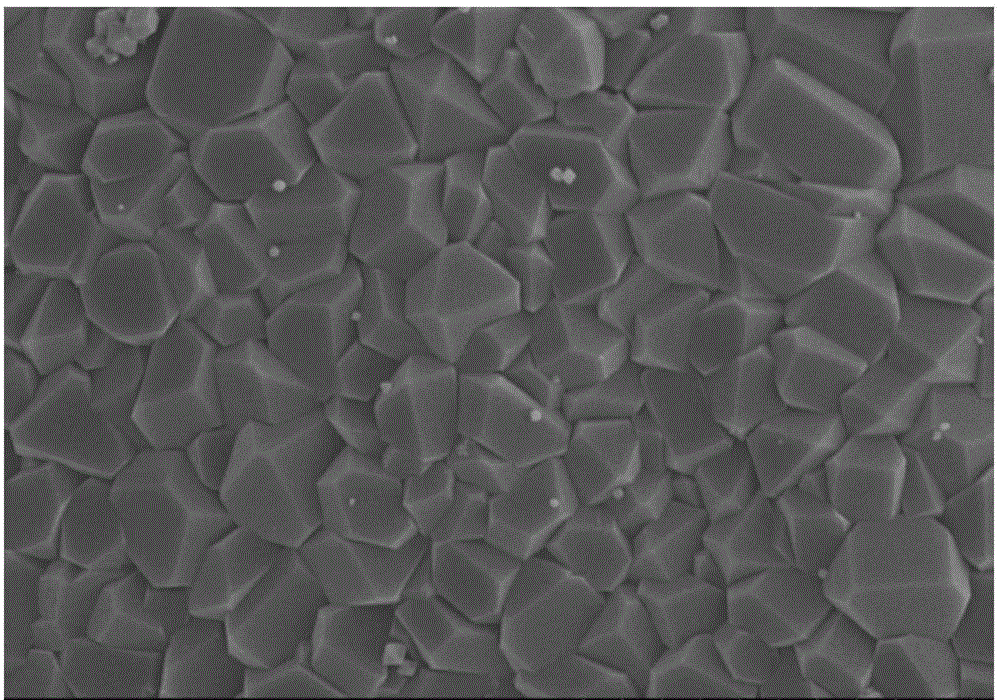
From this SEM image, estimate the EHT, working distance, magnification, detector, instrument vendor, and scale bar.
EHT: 5 kV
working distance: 9.7 mm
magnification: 5 K X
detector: SE(M)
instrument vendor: Hitachi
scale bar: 10000 nm